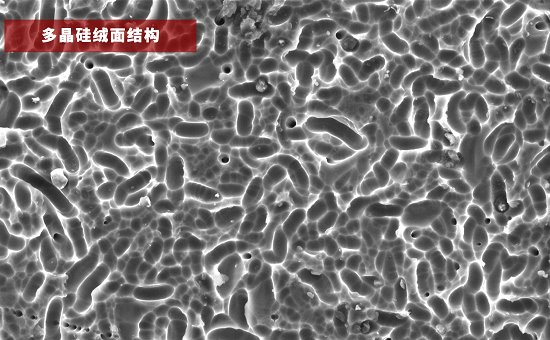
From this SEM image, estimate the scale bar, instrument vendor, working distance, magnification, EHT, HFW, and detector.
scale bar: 30000 nm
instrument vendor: Thermo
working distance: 10.893 mm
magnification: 1 K X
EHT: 15 kV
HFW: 127 µm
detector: SED